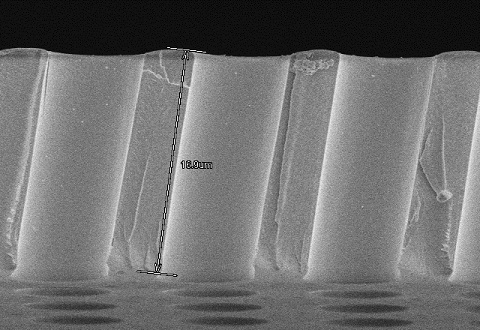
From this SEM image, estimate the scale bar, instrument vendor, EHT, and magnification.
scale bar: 10000 nm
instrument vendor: Hitachi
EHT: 3 kV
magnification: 3.53 K X